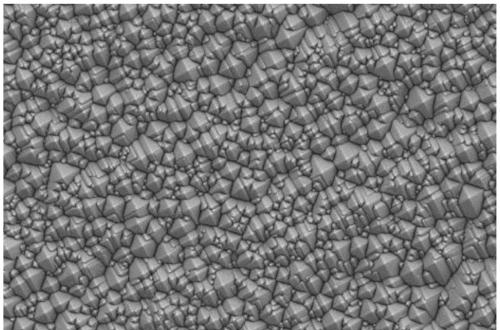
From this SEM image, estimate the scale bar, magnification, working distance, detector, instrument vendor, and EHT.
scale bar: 2000 nm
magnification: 2 K X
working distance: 8.5 mm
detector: SE2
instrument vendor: Zeiss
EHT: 5 kV